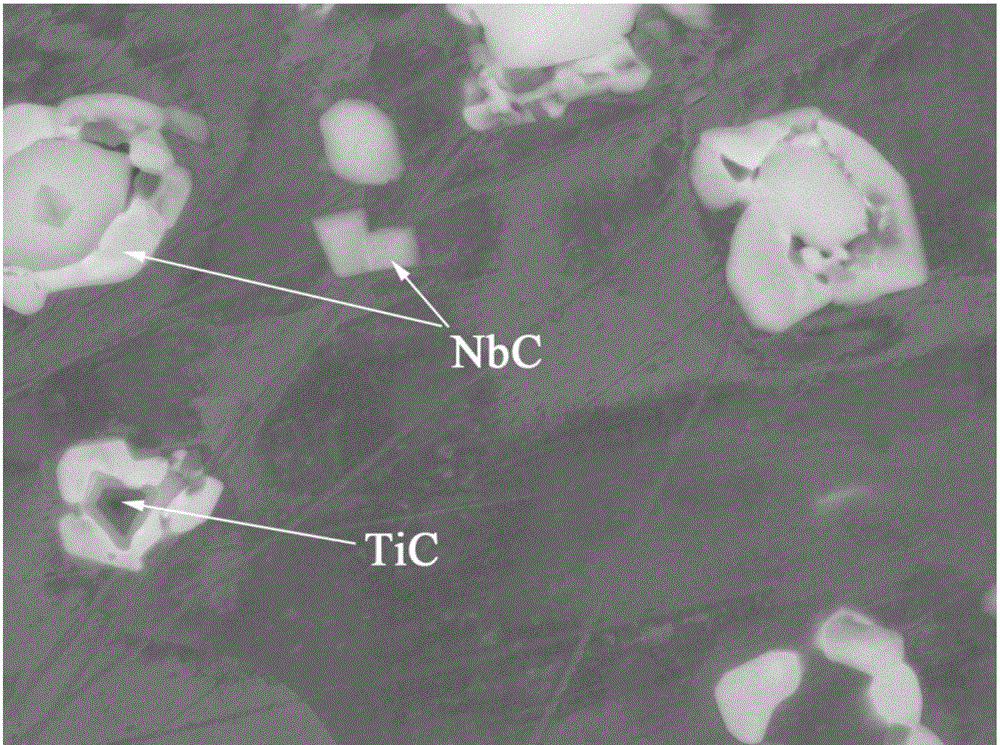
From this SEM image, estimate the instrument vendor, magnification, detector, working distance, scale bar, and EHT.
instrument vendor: JEOL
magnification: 5 K X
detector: COMPO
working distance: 10 mm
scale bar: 1000 nm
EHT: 20 kV